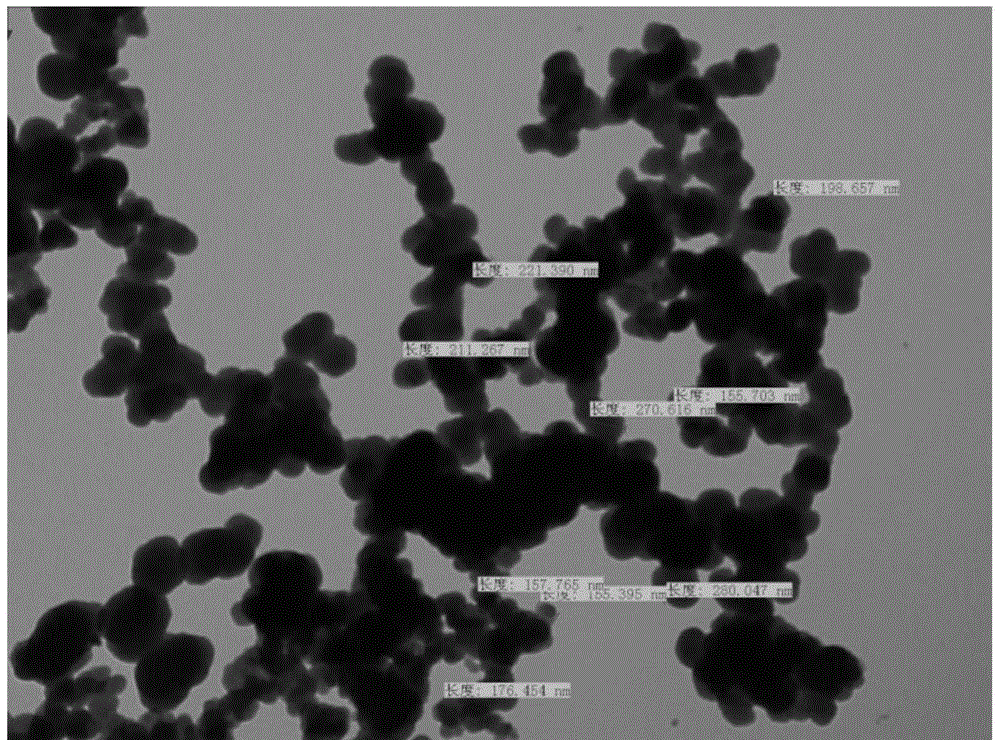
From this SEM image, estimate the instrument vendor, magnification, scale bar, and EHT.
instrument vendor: JEOL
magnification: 27 K X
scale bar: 1000 nm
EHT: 120 kV